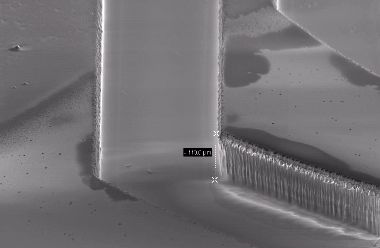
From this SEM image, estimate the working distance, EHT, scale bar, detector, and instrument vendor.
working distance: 19 mm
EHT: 5 kV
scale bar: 30000 nm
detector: SE2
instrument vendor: Zeiss